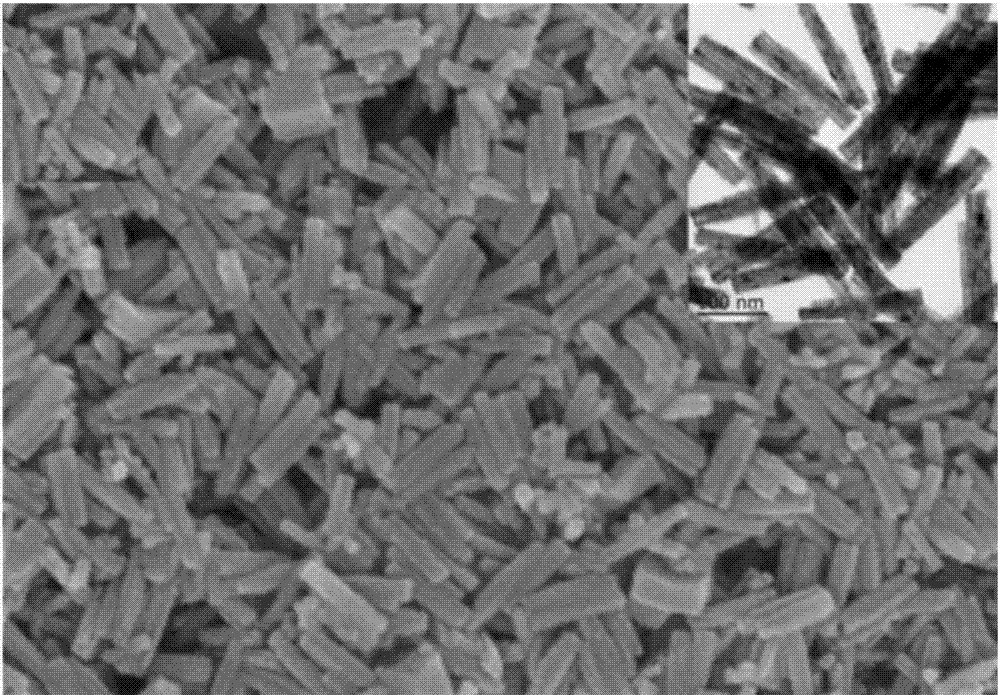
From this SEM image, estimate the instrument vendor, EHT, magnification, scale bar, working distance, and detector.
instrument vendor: Hitachi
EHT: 15 kV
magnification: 60 K X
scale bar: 500 nm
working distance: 7.4 mm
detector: SE(M)